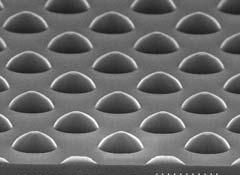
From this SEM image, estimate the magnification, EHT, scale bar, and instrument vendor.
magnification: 7 K X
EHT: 20 kV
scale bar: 4290 nm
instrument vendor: JEOL